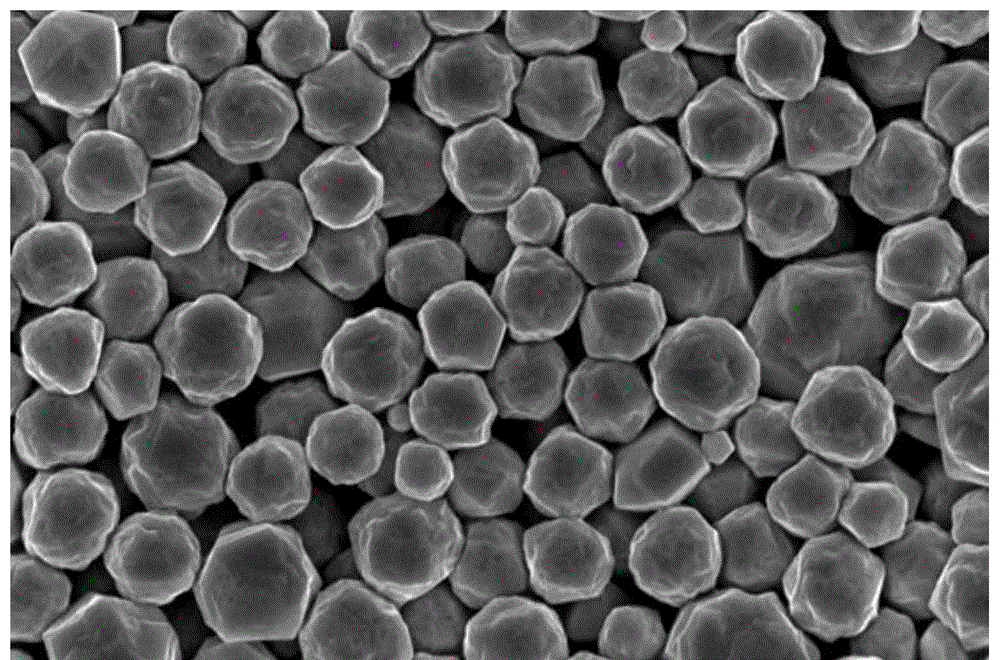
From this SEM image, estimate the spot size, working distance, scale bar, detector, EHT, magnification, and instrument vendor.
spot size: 3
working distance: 5.7 mm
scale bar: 3000 nm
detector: TLD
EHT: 10 kV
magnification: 50 K X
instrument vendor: FEI